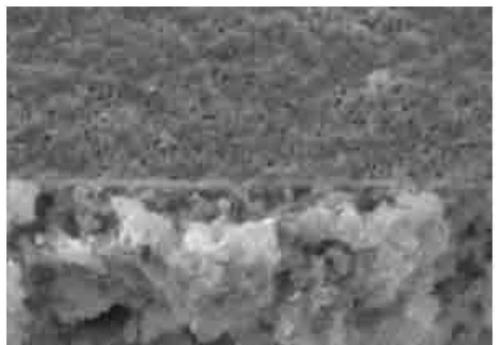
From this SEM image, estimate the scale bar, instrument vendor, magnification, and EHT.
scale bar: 10000 nm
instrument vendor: other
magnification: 2 K X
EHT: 25 kV